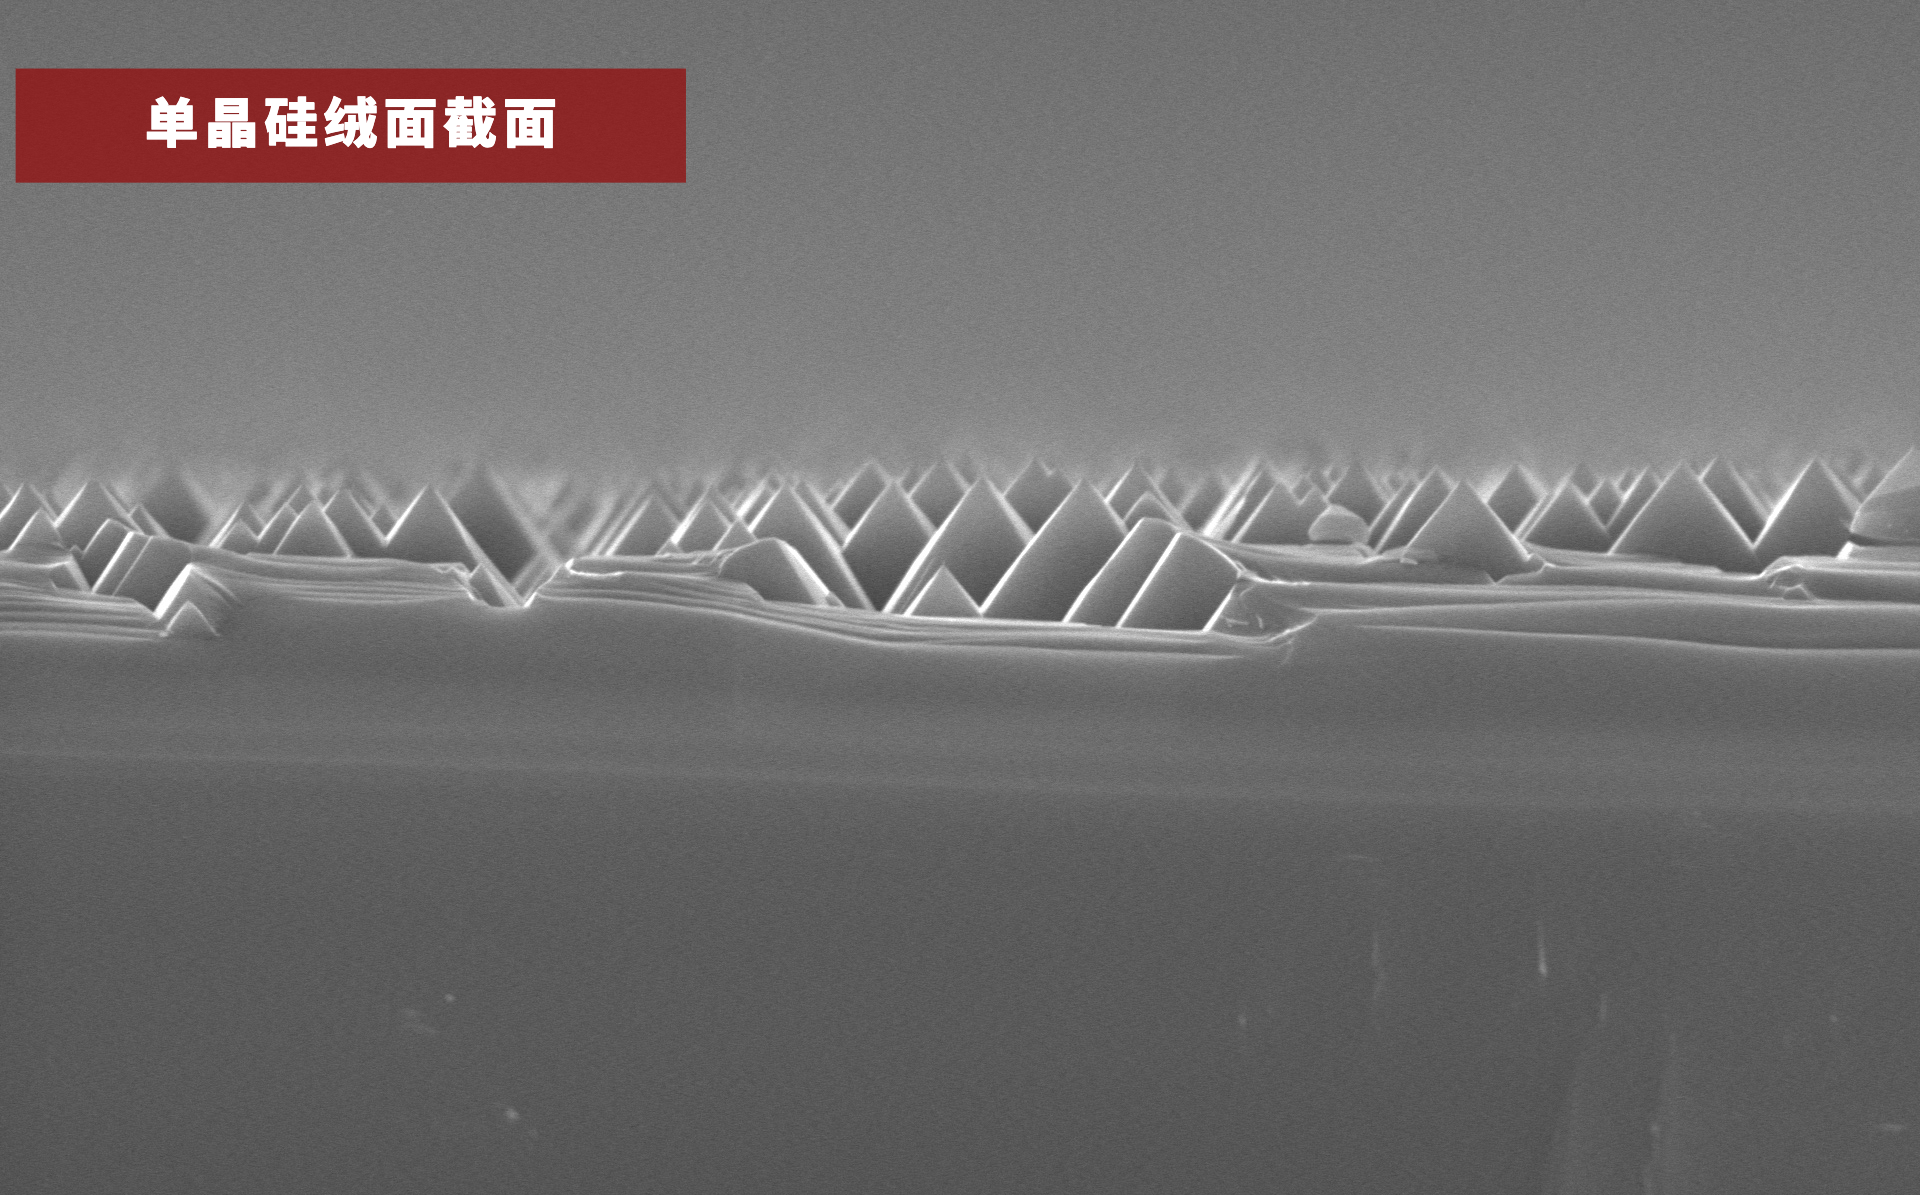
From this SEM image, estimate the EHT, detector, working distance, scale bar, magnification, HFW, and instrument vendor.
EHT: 10 kV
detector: SED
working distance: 7.385 mm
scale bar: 8000 nm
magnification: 5 K X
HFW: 25.4 µm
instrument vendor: other